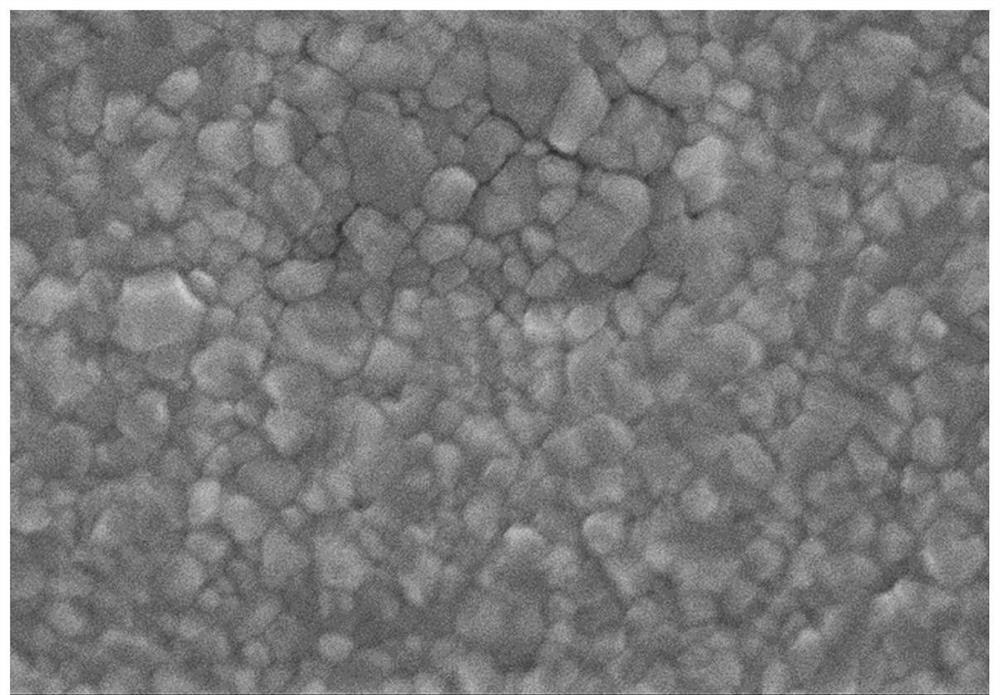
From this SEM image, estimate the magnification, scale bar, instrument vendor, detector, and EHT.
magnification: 30 K X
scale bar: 1000 nm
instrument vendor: Hitachi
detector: SE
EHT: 5 kV